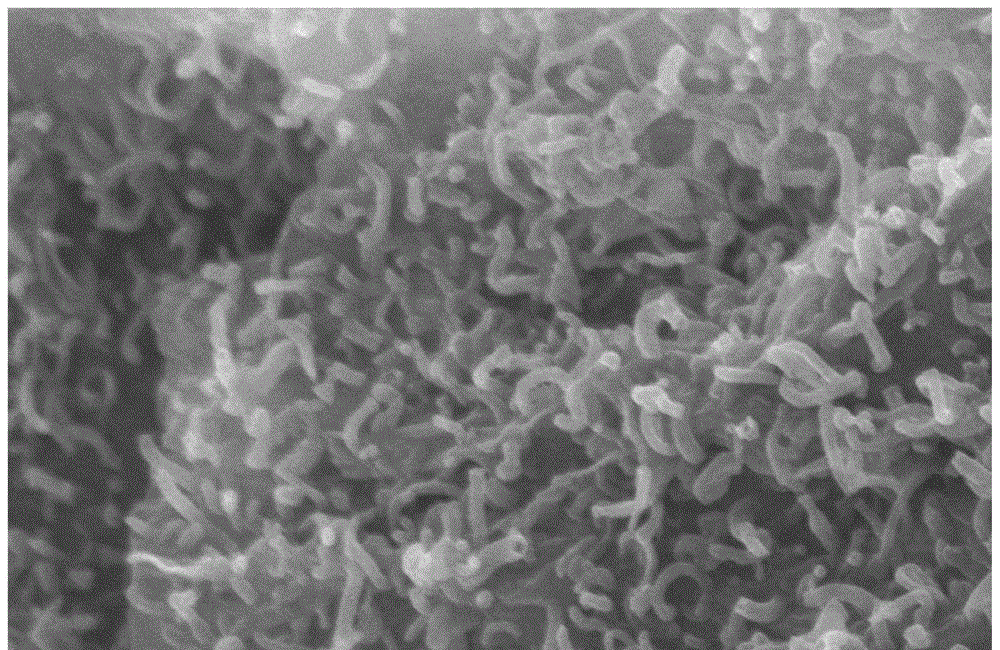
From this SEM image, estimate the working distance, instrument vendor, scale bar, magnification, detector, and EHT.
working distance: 10.6 mm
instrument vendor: Hitachi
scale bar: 1000 nm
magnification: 50 K X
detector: SE(M)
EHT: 5 kV